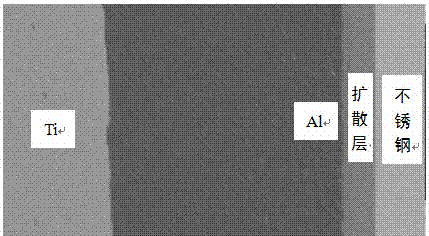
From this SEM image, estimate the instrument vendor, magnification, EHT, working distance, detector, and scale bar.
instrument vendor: FEI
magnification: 0.4 K X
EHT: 20 kV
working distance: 10 mm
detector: BSE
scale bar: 50000 nm